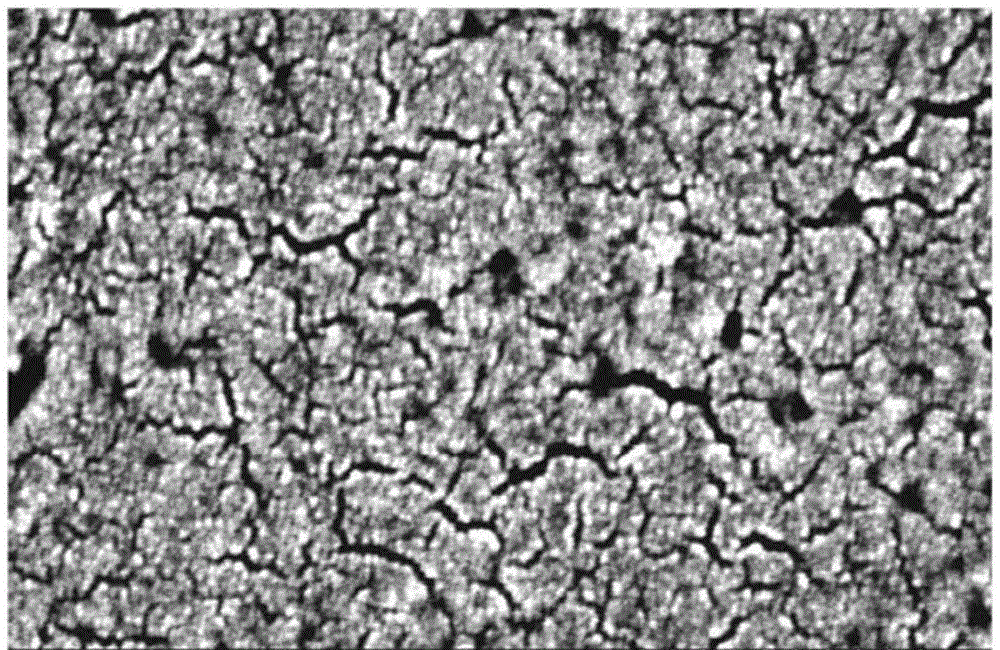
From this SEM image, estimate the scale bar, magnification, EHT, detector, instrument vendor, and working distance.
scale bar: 200 nm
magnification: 200 K X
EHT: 5 kV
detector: SE(M)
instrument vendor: Hitachi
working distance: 8.6 mm